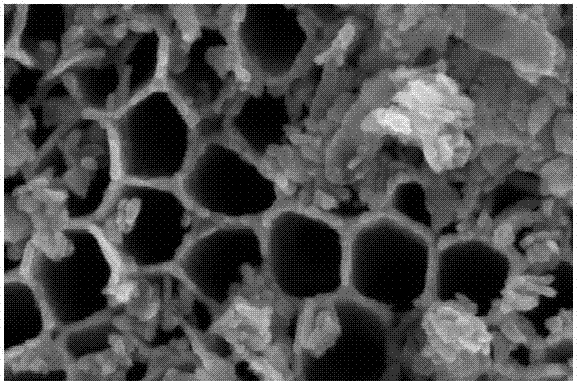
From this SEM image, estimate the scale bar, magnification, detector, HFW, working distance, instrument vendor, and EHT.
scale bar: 300 nm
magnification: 600.024 K X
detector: TLD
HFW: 0.691 µm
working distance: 3.8 mm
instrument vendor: FEI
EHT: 5 kV